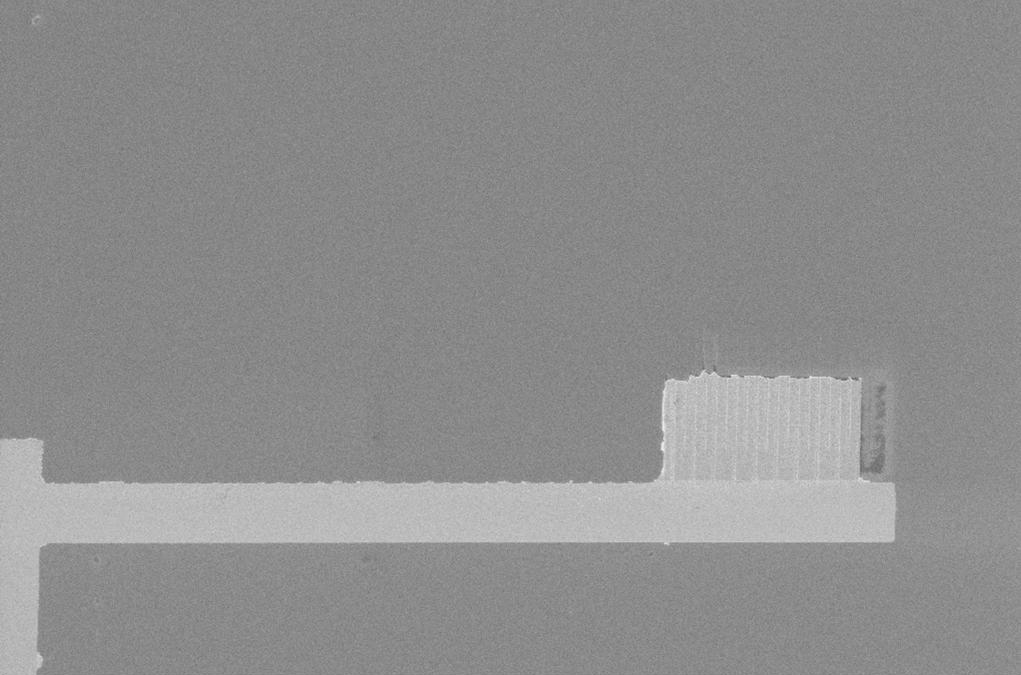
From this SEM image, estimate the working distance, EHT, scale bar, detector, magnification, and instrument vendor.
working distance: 9 mm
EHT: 5 kV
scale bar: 10000 nm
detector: InLens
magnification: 3.43 K X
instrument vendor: Zeiss